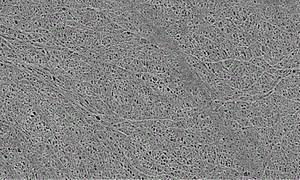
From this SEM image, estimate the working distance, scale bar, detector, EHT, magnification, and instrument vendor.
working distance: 9.7 mm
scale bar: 10000 nm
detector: SE(UL)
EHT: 1 kV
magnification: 3 K X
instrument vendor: Hitachi